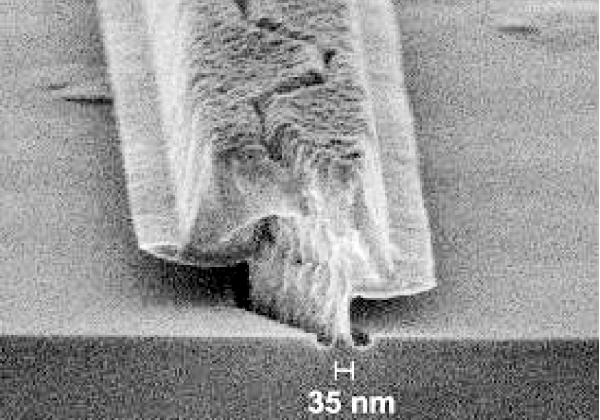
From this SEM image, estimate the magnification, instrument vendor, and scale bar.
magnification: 150 K X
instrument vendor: Zeiss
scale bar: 200 nm